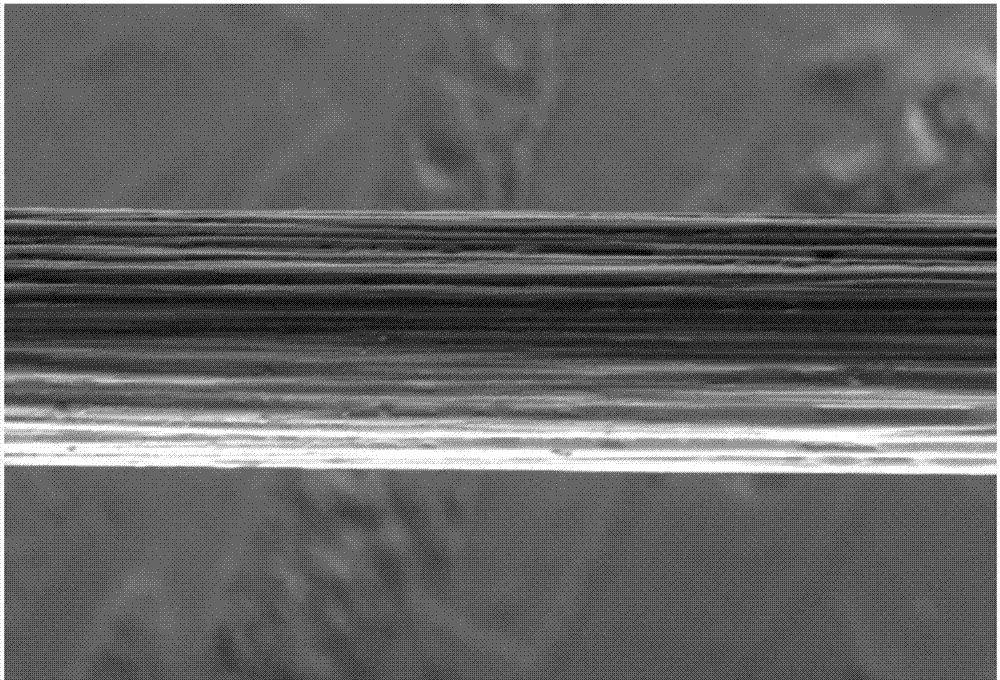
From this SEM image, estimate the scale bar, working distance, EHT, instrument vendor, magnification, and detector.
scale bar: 4000 nm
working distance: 5.6 mm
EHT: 3 kV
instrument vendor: FEI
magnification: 10 K X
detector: ETD